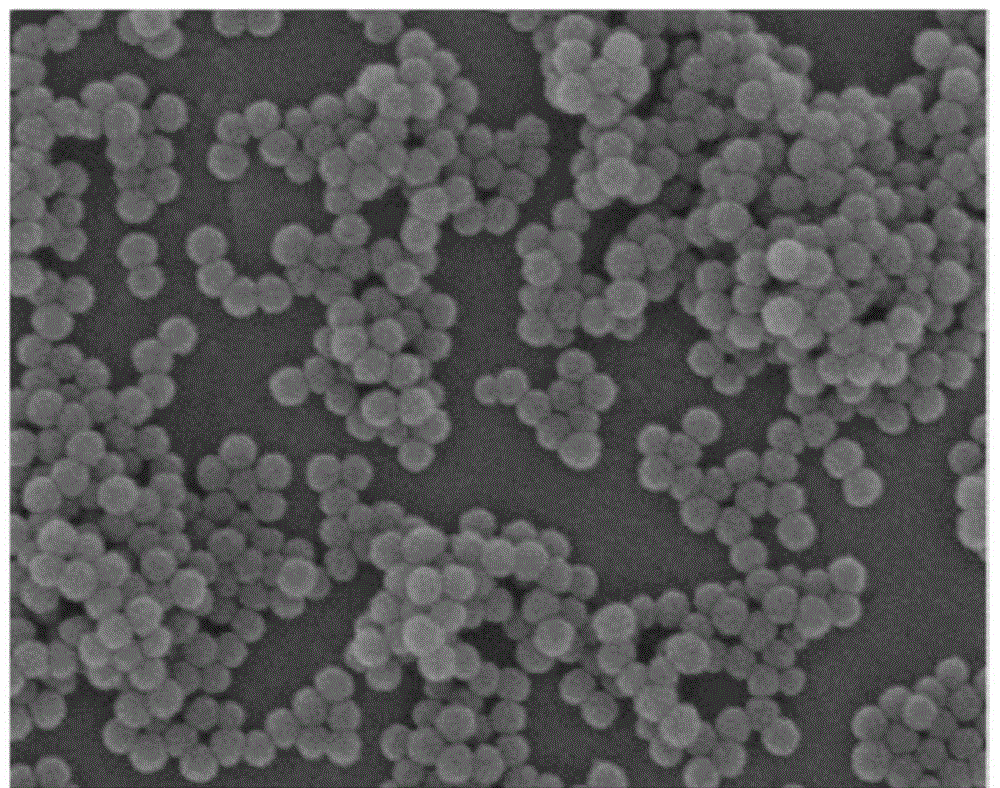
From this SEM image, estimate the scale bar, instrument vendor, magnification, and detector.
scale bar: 500 nm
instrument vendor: Hitachi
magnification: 100 K X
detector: SE(U)